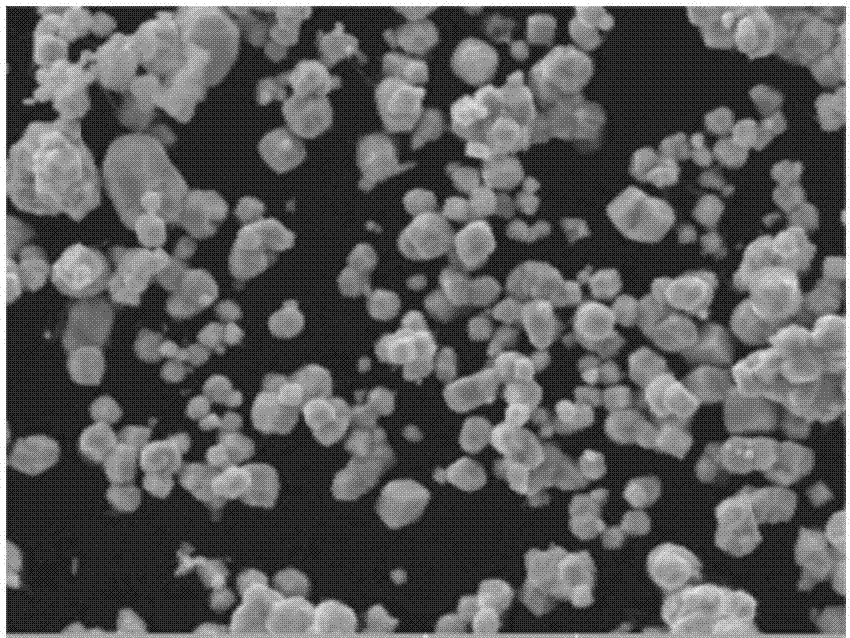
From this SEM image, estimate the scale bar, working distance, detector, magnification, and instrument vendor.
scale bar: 5000 nm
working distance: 8.12 mm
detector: SE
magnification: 10 K X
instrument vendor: Tescan